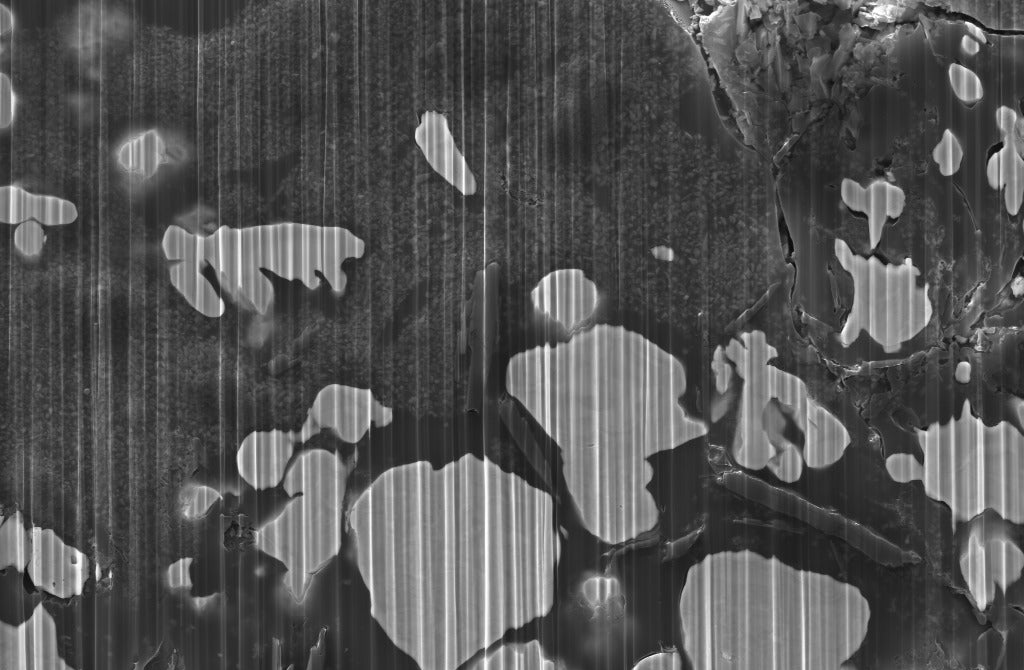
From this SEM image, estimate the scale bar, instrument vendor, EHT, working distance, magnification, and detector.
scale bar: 2000 nm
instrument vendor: Zeiss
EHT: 10 kV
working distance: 10.1 mm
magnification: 3 K X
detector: SE2